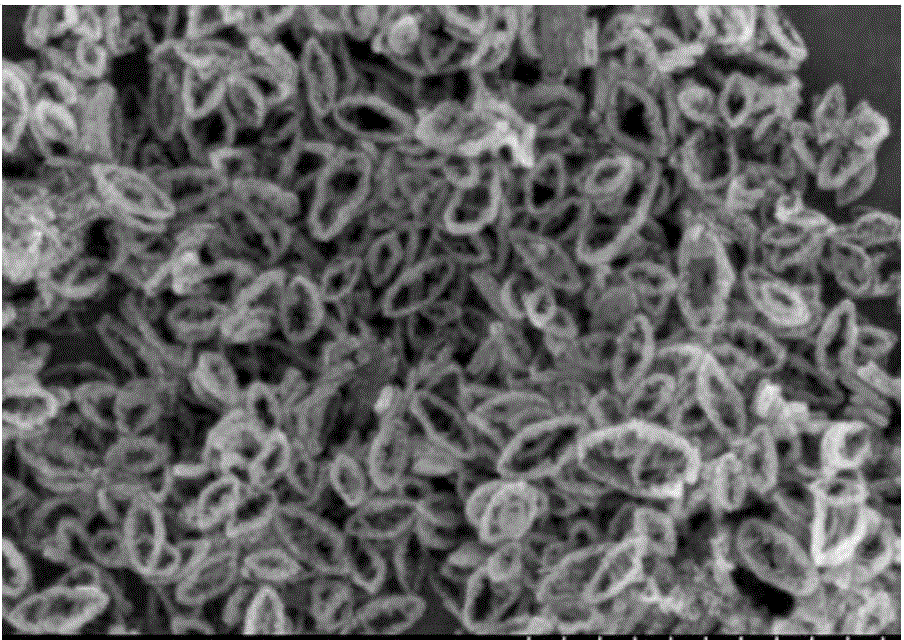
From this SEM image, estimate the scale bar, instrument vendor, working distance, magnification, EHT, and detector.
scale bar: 1000 nm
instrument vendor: Hitachi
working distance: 7.4 mm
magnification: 50 K X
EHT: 5 kV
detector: SE(M)